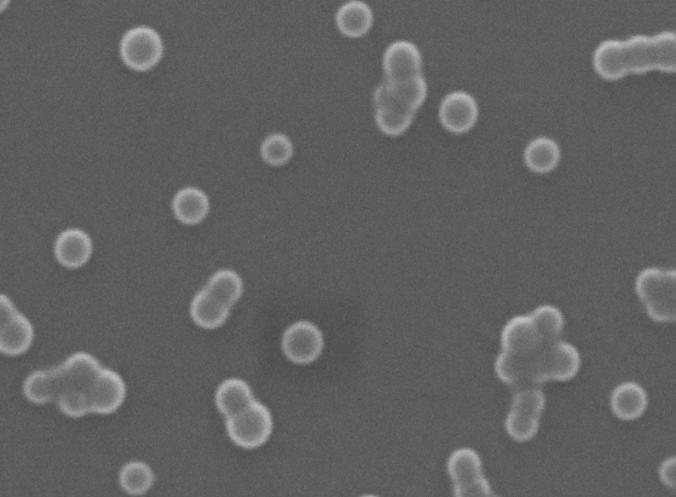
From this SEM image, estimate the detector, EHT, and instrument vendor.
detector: SE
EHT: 20 kV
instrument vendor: Tescan